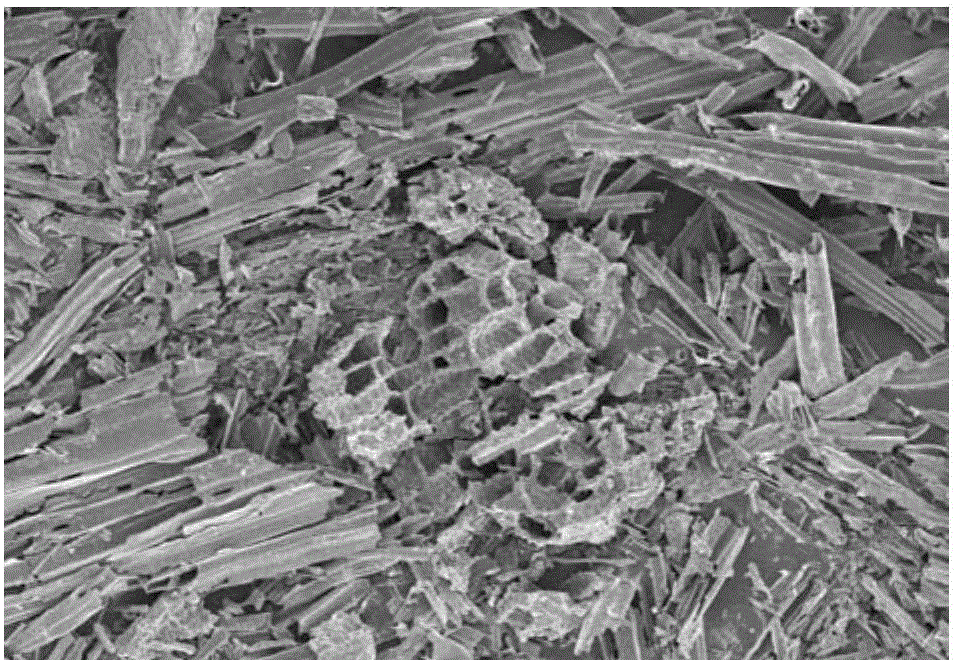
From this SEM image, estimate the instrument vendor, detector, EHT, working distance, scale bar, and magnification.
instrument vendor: Hitachi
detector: SE(M)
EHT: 3 kV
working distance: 8.8 mm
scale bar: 100000 nm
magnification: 0.5 K X